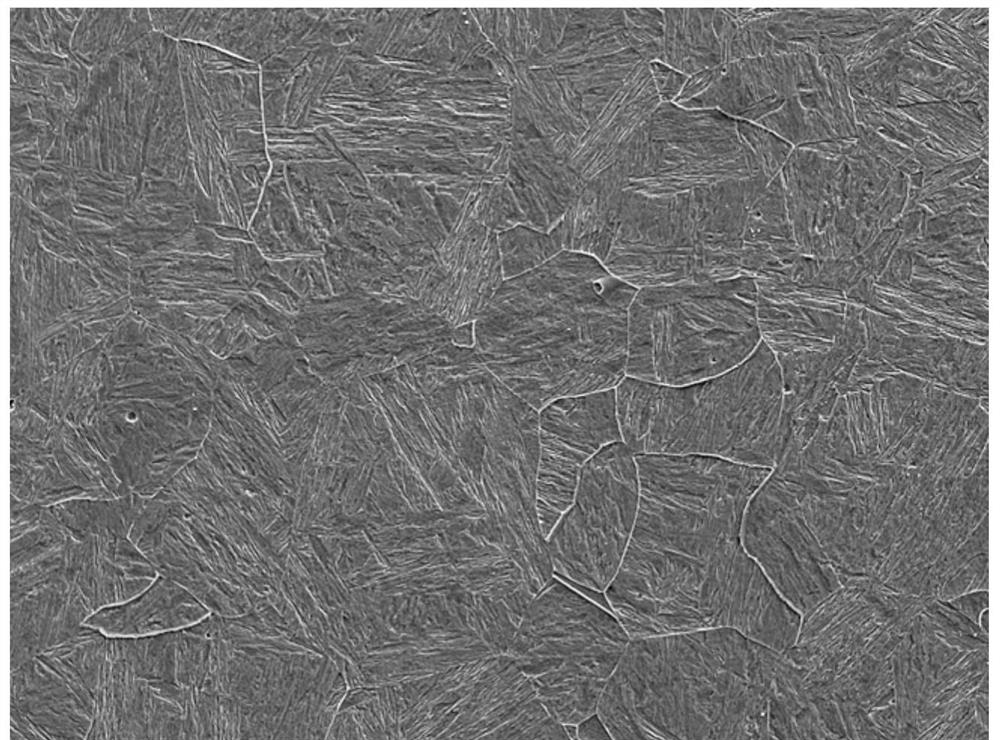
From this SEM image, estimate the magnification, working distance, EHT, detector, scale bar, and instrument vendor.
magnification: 0.5 K X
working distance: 10 mm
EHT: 15 kV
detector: LED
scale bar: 10000 nm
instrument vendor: JEOL